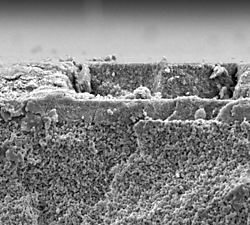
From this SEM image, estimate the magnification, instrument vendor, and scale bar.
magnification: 5 K X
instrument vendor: JEOL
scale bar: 5000 nm